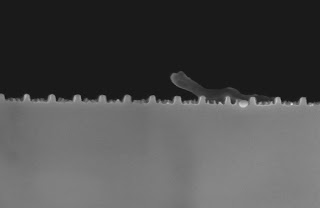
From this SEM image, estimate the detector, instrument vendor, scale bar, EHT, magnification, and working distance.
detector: InLens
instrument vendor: Zeiss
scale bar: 200 nm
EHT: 3 kV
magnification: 98.11 K X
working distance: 5 mm